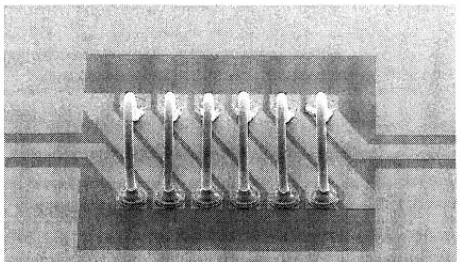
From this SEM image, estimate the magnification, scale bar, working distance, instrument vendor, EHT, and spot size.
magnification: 0.2 K X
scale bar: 200000 nm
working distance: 13 mm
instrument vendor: FEI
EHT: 5 kV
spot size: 3